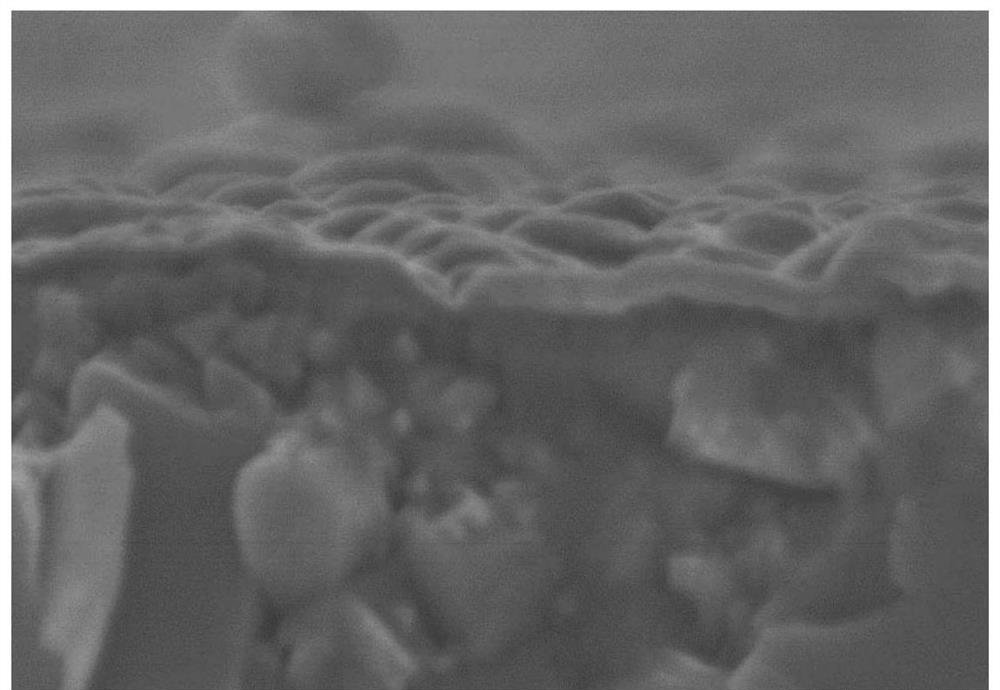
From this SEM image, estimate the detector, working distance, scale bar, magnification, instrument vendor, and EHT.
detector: SE(U)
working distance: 7.3 mm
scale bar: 500 nm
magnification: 80 K X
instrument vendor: Hitachi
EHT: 5 kV